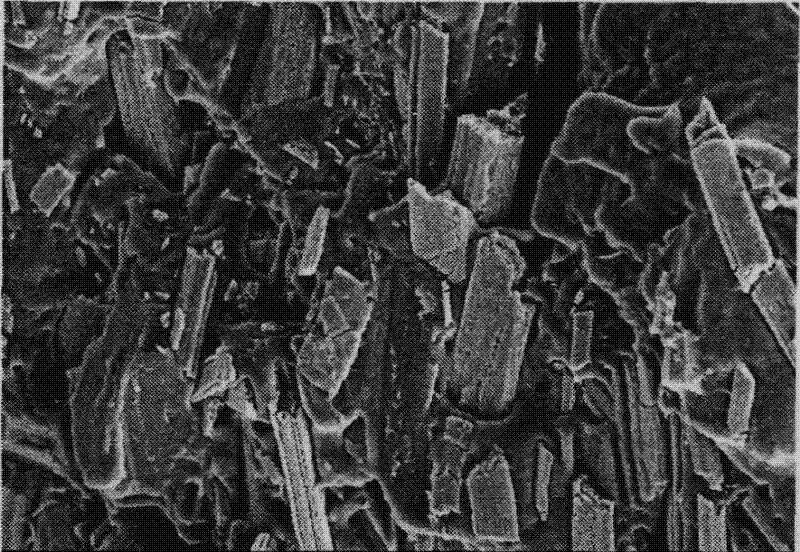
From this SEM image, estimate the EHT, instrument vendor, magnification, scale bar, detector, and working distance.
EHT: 10 kV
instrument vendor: Hitachi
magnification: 1 K X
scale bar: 50000 nm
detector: SE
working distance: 16.3 mm